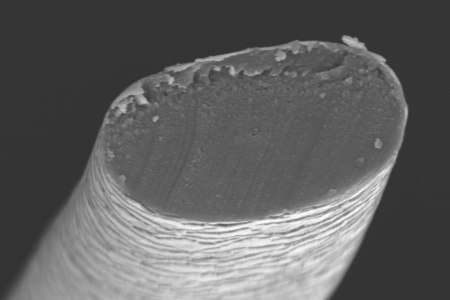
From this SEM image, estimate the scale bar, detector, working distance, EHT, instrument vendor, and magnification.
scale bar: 50000 nm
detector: SE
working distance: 15.2 mm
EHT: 15 kV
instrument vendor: JEOL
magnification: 1 K X